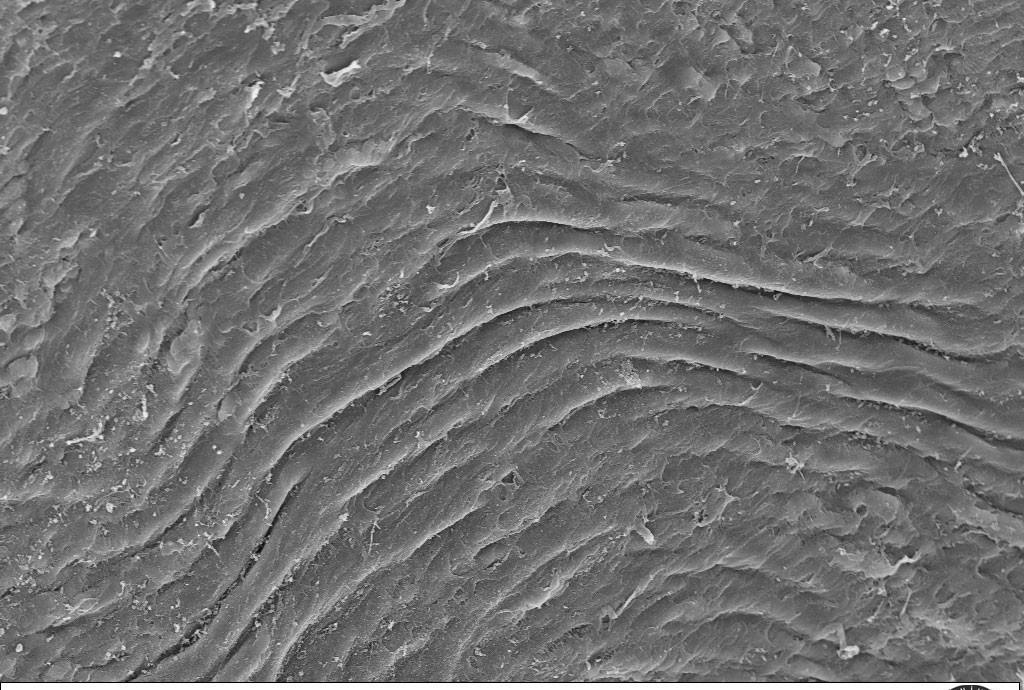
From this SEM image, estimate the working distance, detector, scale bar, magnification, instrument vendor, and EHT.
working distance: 24 mm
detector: SE1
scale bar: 20000 nm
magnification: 0.65 K X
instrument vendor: Zeiss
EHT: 20 kV